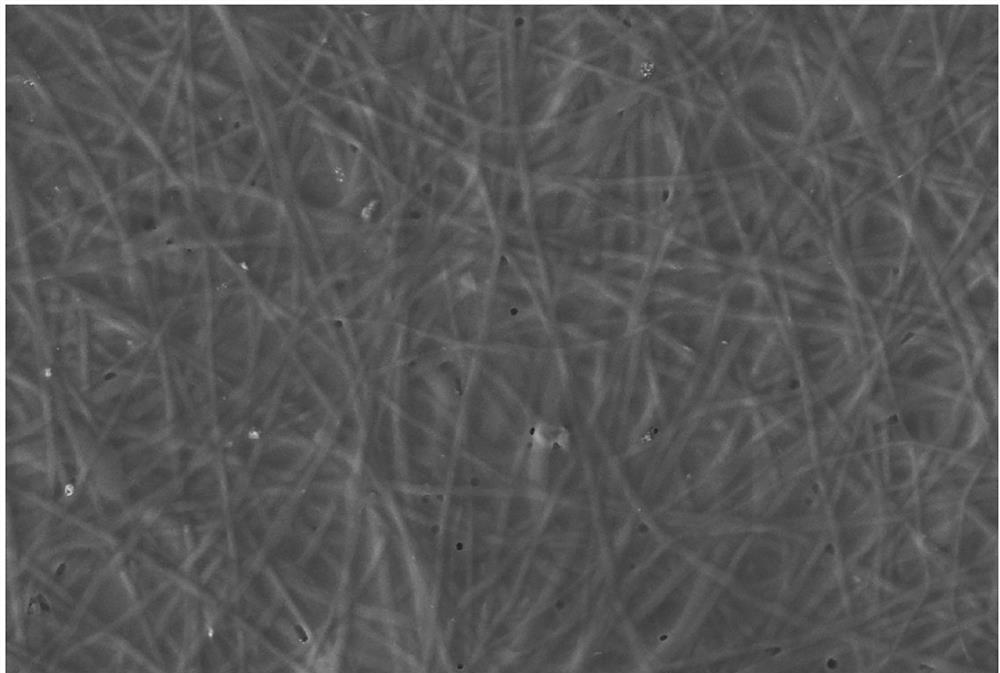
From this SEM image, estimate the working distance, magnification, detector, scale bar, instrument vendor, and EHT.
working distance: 6.1 mm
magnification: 5 K X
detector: SE2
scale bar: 1000 nm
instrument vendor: Zeiss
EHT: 3 kV